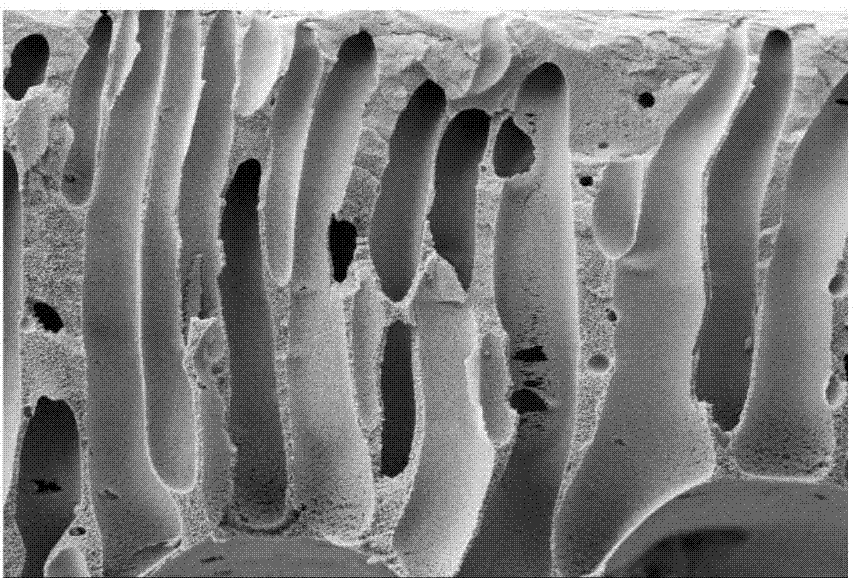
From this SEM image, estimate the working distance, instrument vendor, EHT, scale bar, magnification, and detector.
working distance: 5.8 mm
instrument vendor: Zeiss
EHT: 6 kV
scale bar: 10000 nm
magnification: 0.8 K X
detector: SE2